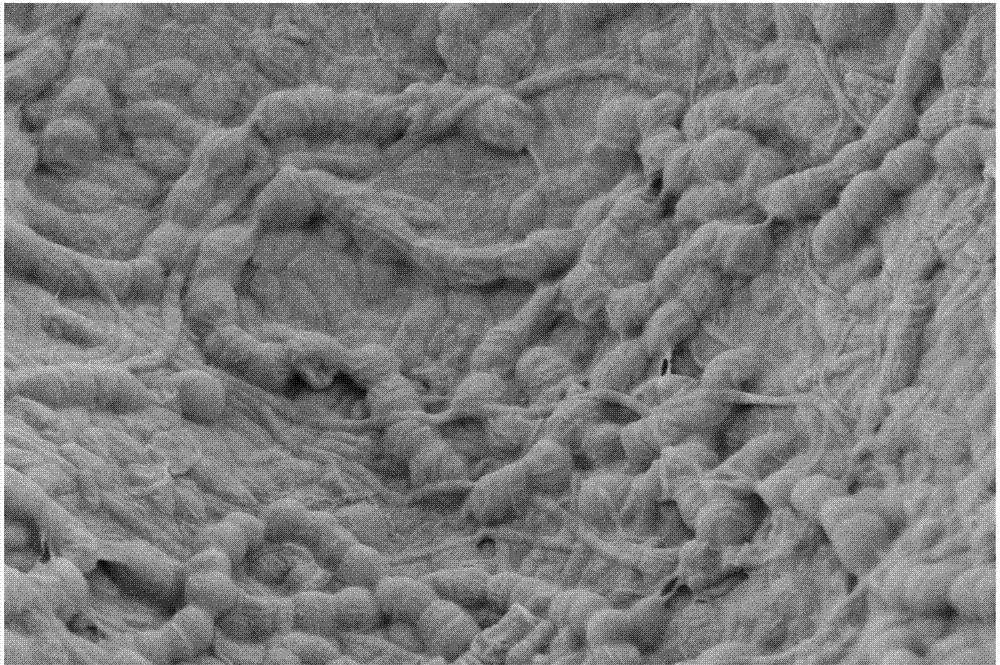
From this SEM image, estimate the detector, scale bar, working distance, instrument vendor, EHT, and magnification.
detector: MPSE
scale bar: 2000 nm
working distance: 4 mm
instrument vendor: Zeiss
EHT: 5 kV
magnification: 5 K X